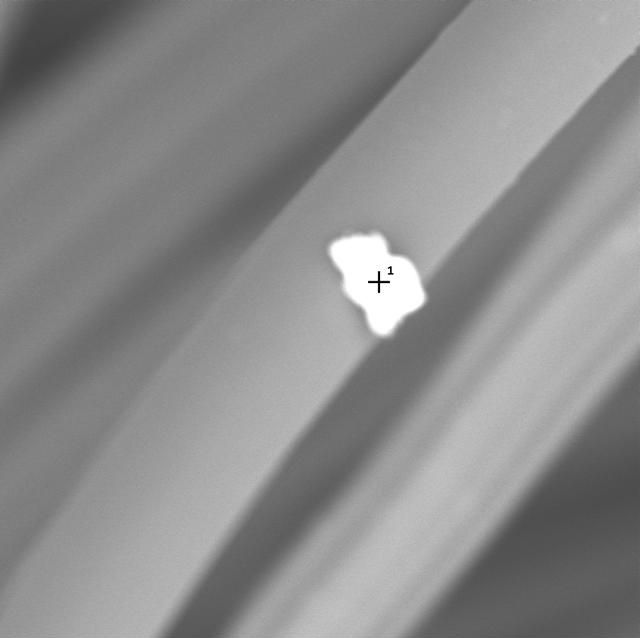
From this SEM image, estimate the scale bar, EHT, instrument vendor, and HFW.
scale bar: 10000 nm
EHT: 15 kV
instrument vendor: other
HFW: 53.7 µm